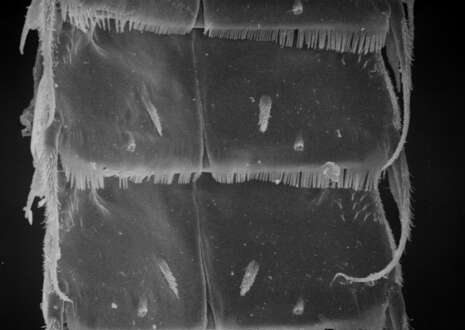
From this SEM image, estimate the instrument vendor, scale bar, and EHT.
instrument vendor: JEOL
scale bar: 20000 nm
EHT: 10 kV